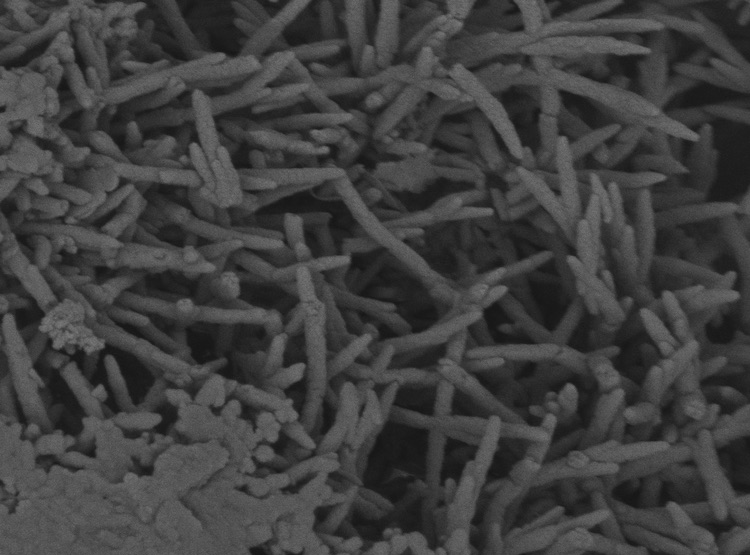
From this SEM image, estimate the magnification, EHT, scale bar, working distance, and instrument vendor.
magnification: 26.518 K X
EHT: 3 kV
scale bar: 2000 nm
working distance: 4.8 mm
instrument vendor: FEI